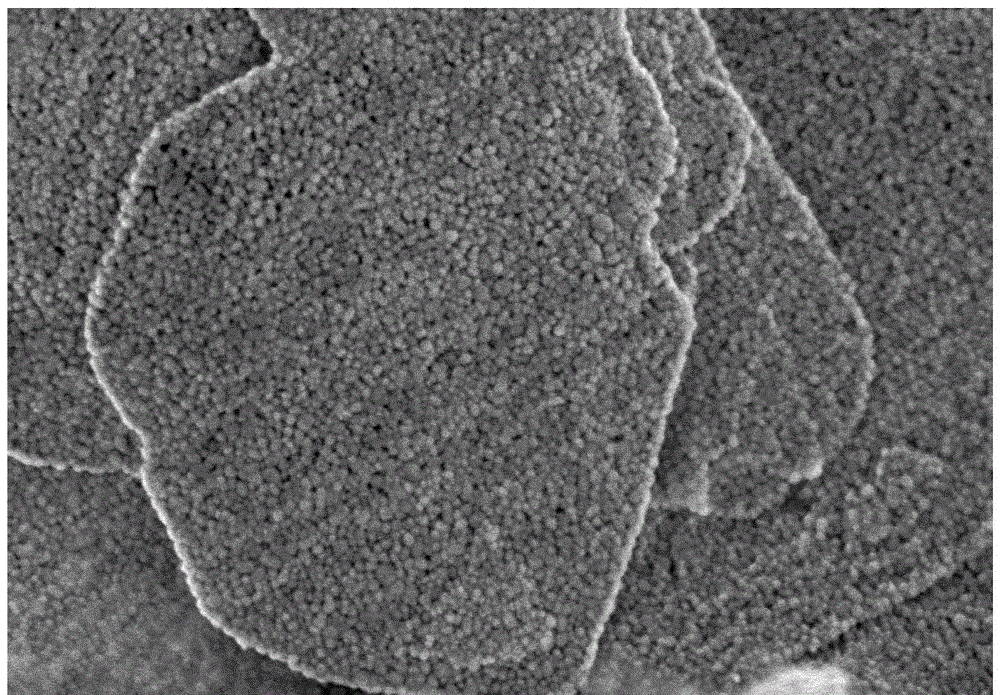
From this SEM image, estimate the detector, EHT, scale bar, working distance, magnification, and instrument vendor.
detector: SE+BSE(TU)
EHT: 1 kV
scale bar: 500 nm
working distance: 1.8 mm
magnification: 100 K X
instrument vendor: Hitachi